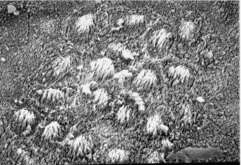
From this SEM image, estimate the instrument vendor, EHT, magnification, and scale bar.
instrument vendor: JEOL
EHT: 20 kV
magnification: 1.2 K X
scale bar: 10000 nm